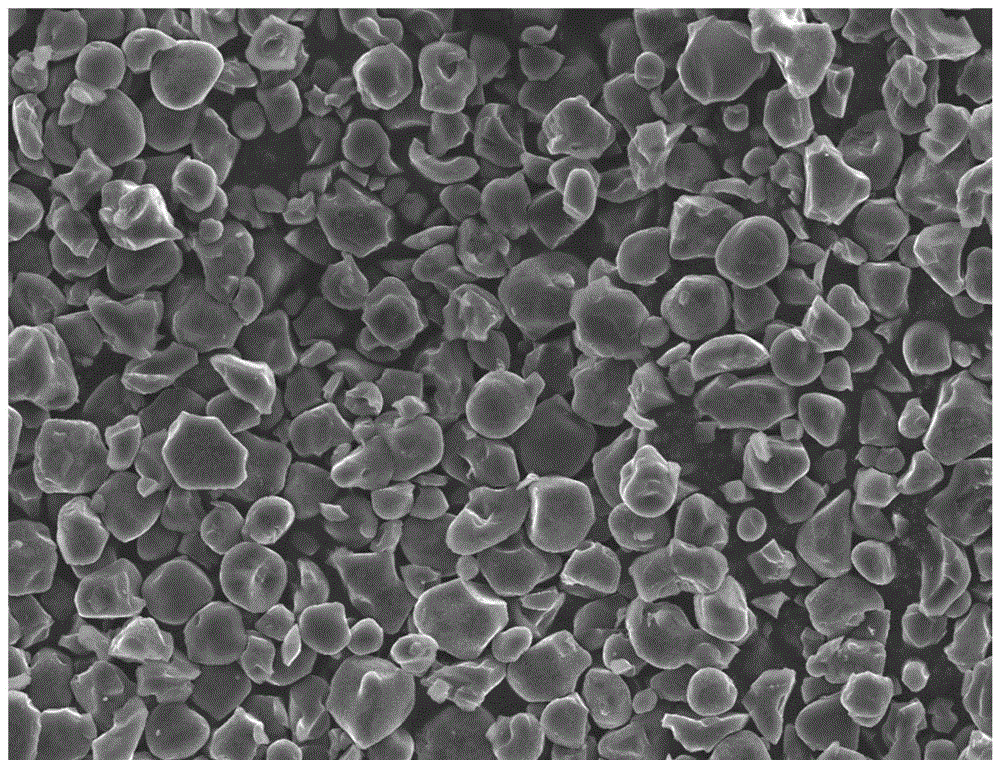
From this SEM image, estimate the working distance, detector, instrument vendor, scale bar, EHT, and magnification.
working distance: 10.3 mm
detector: SED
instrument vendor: JEOL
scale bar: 10000 nm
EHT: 10 kV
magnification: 1 K X